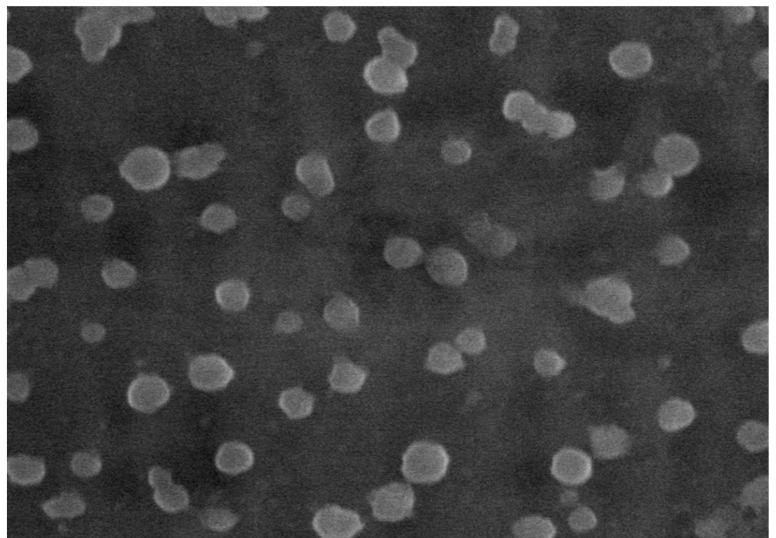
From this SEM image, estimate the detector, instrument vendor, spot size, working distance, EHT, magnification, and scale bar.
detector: LFD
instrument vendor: FEI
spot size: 3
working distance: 10.8 mm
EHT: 20 kV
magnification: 100 K X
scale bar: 500 nm